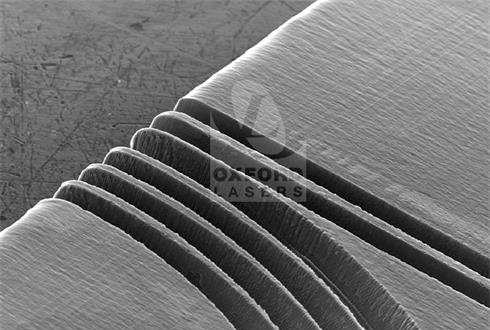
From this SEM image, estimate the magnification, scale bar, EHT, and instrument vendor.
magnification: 0.075 K X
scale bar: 100000 nm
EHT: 30 kV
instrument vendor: JEOL